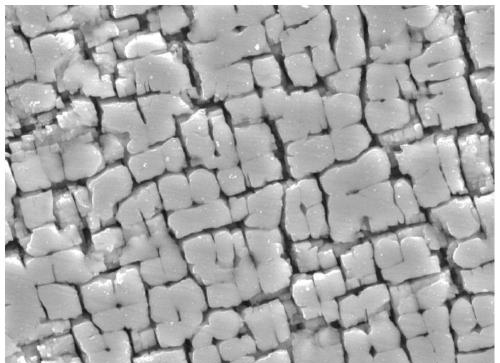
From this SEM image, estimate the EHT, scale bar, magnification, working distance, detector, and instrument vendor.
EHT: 15 kV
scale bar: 1000 nm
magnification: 10 K X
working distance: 9.6 mm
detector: LED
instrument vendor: JEOL